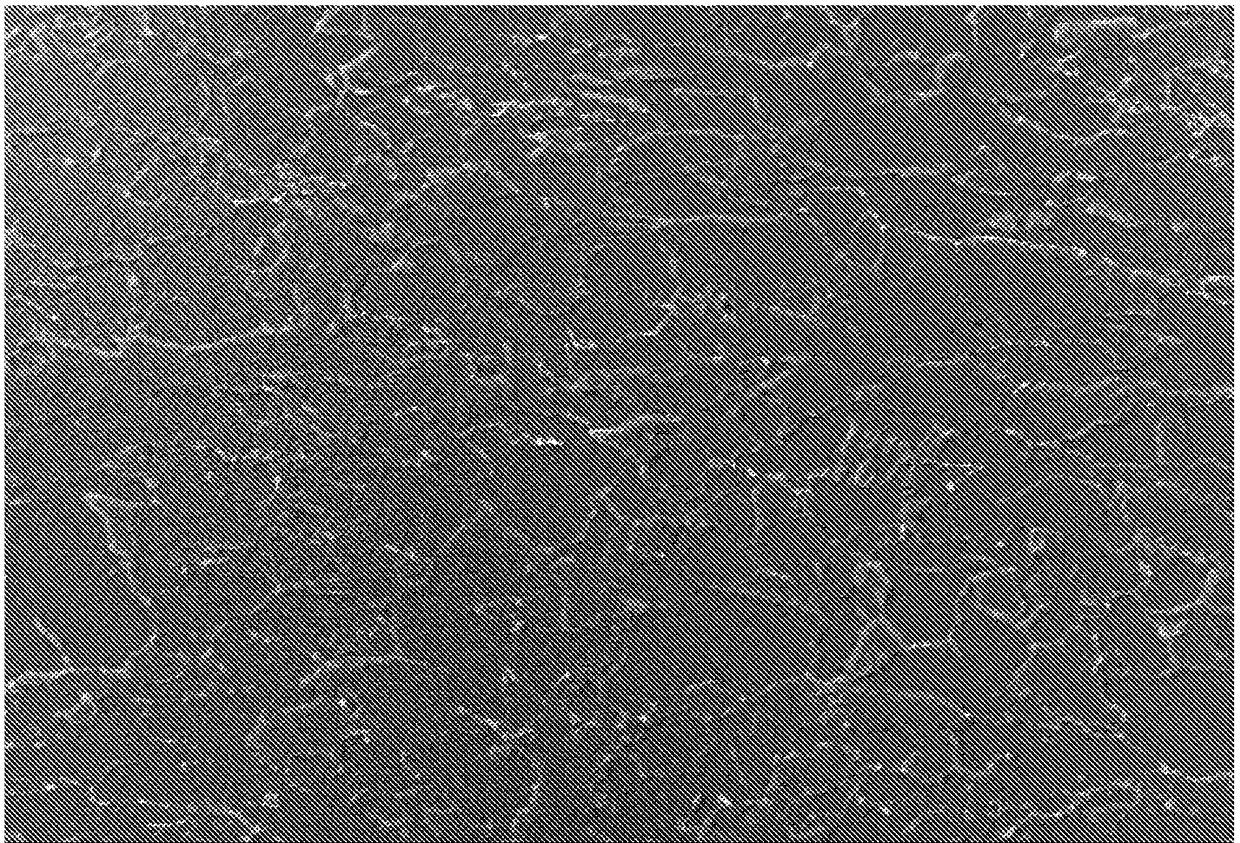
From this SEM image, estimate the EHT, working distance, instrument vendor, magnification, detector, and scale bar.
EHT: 20 kV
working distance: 10 mm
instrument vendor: JEOL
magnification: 1 K X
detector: SEI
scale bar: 10000 nm